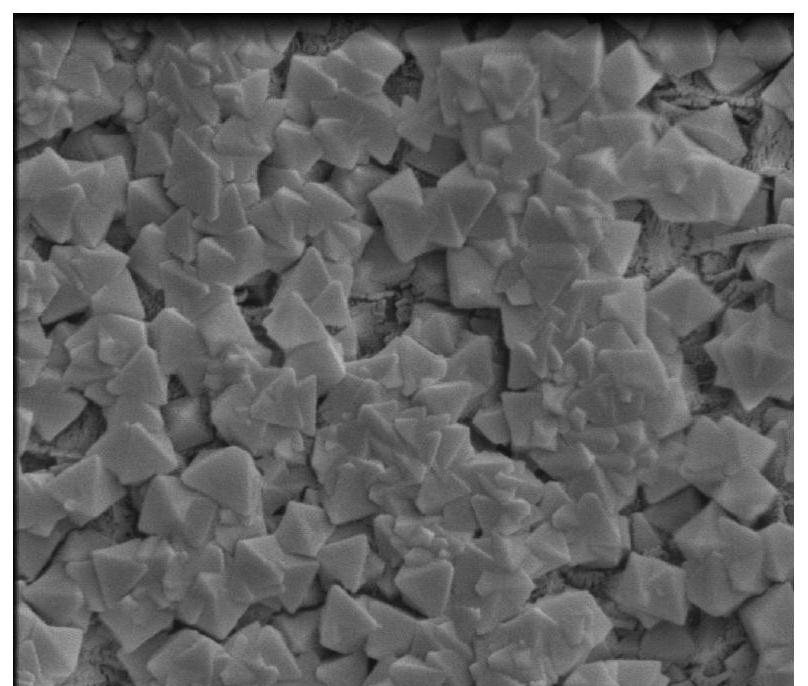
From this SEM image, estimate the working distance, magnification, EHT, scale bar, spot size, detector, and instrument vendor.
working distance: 7.8 mm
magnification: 40 K X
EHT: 5 kV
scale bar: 2000 nm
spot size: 3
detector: ETD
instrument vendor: FEI